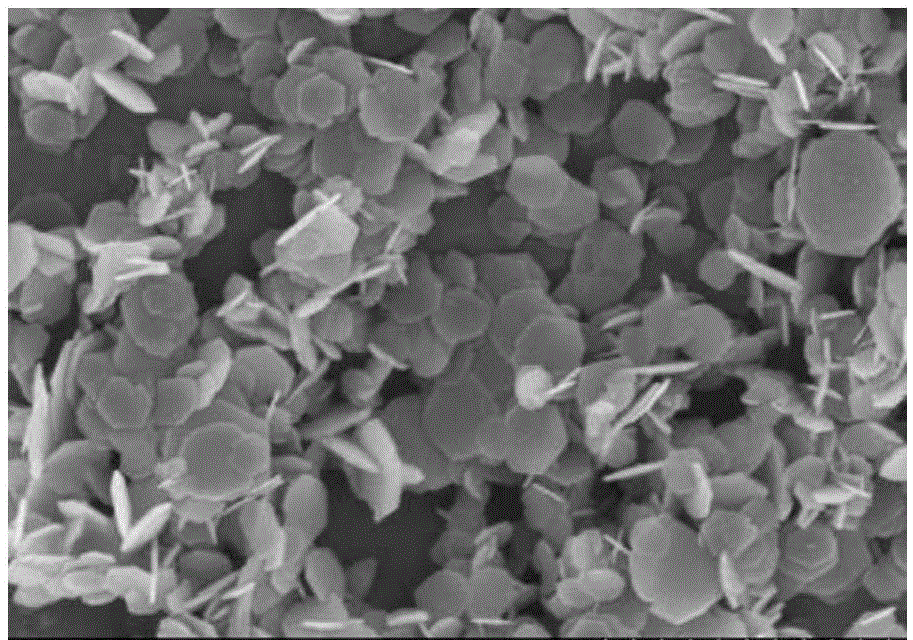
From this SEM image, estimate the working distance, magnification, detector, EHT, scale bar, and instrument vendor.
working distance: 6.9 mm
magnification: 20 K X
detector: SE(M)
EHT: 5 kV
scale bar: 2000 nm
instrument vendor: Hitachi